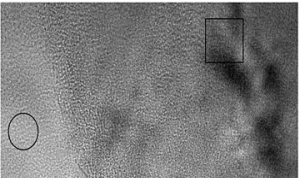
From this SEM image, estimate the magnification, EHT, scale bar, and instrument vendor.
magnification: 800 K X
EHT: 200 kV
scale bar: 20 nm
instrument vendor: JEOL